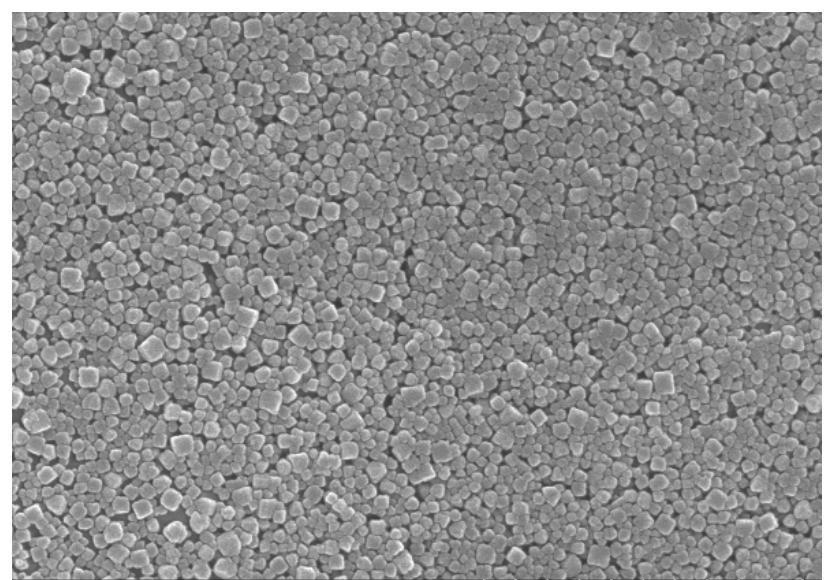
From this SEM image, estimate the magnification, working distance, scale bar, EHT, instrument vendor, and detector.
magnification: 50 K X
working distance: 8.8 mm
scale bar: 1000 nm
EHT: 10 kV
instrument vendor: Hitachi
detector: SE(U)